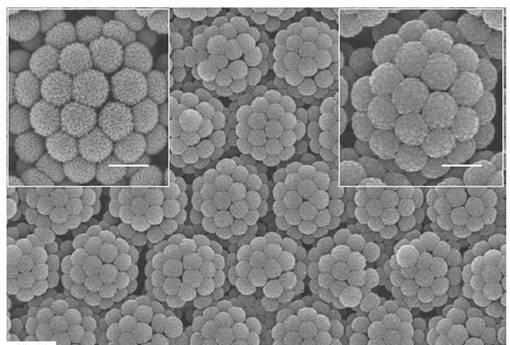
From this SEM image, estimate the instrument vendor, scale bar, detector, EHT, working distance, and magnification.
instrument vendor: Hitachi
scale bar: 5000 nm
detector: SE(M)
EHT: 15 kV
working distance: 9.9 mm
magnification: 10 K X